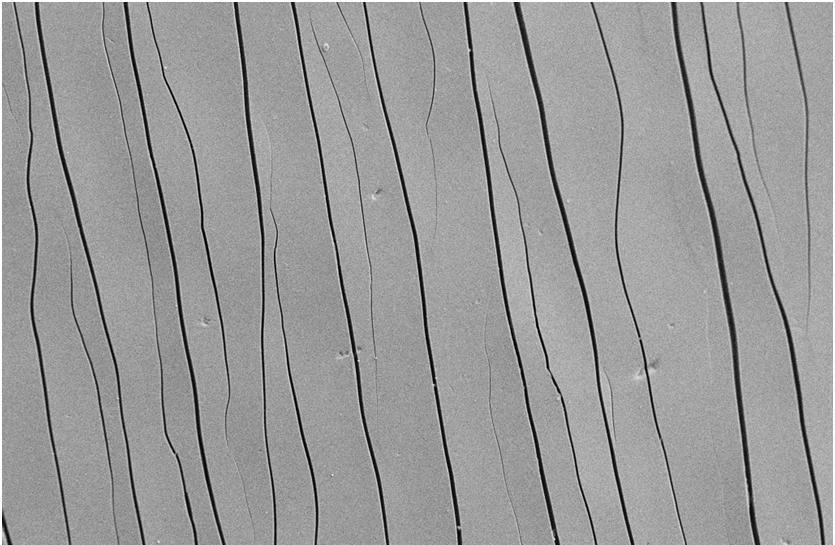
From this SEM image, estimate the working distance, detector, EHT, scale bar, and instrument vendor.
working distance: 5 mm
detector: SE2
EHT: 10 kV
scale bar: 10000 nm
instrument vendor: Zeiss